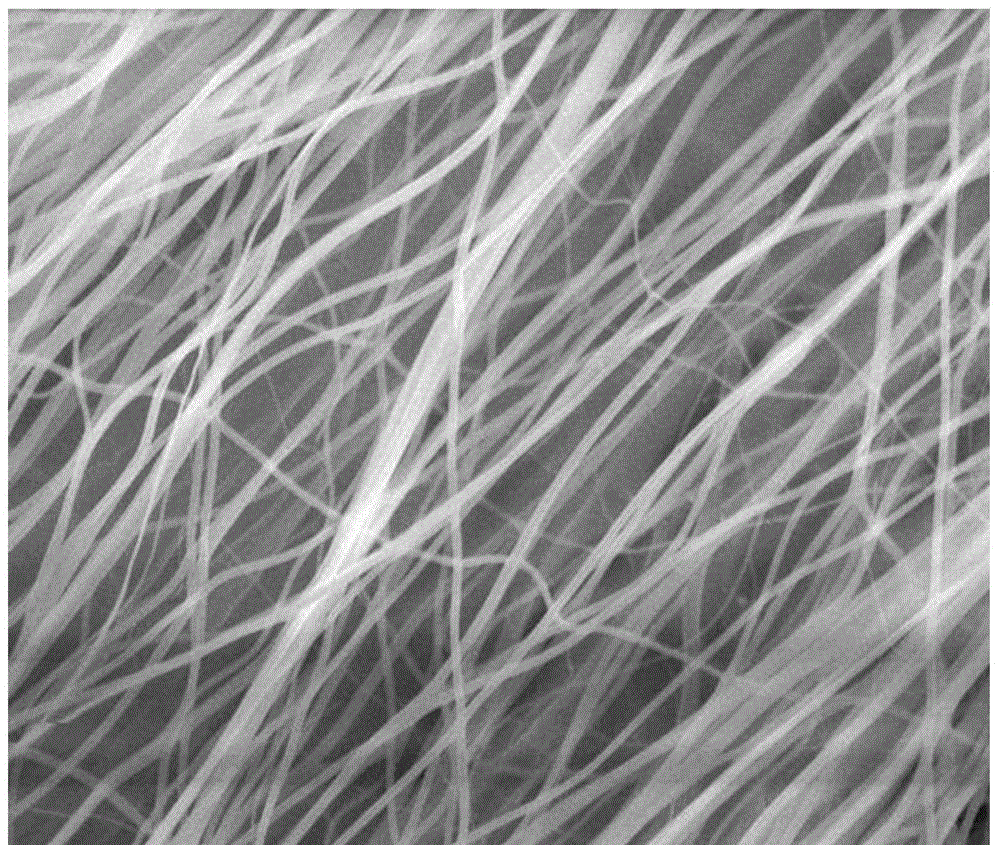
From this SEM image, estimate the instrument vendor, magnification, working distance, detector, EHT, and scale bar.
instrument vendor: JEOL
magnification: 20 K X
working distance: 9.4 mm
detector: SEI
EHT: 15 kV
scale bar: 1000 nm